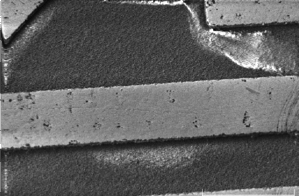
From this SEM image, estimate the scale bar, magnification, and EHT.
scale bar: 500000 nm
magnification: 0.0619 K X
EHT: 5 kV